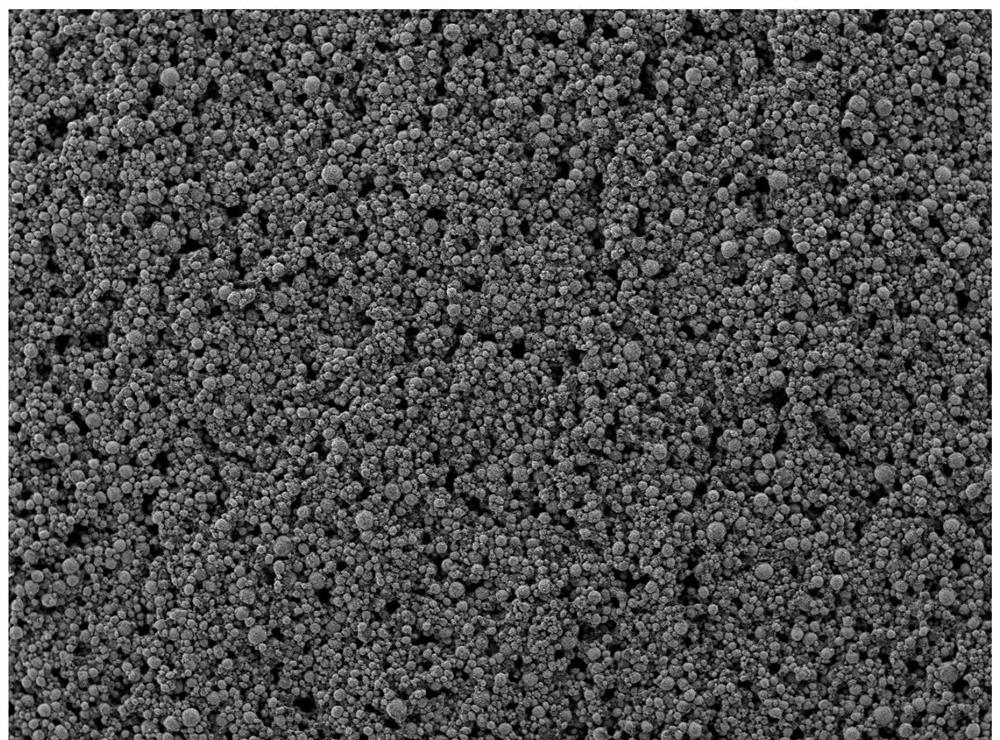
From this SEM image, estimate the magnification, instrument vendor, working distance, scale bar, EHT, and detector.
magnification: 0.1 K X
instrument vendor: JEOL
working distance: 7.9 mm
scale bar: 100000 nm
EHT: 10 kV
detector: LED